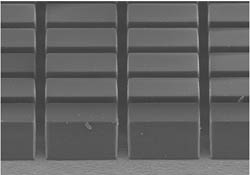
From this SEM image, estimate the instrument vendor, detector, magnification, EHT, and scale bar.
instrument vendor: Hitachi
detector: SE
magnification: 0.2 K X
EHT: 15 kV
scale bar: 200000 nm